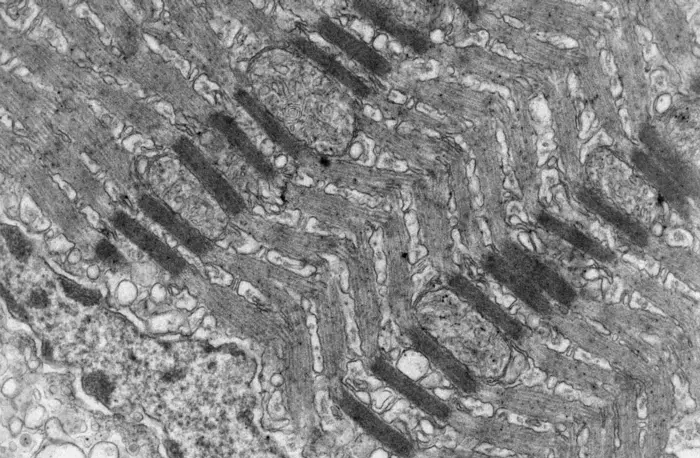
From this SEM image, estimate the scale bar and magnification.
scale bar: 1000 nm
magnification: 10 K X